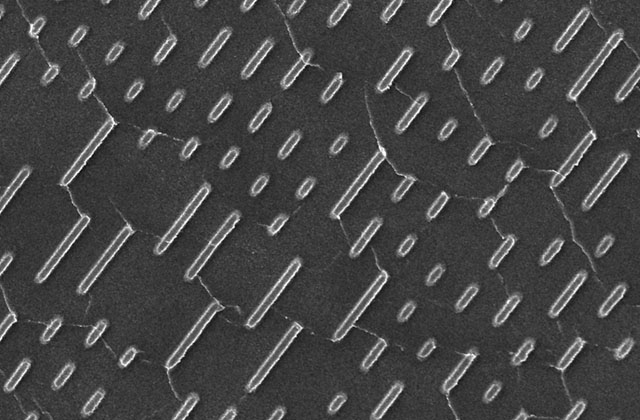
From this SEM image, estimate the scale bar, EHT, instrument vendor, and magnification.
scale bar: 5000 nm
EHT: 15 kV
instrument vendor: JEOL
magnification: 5 K X